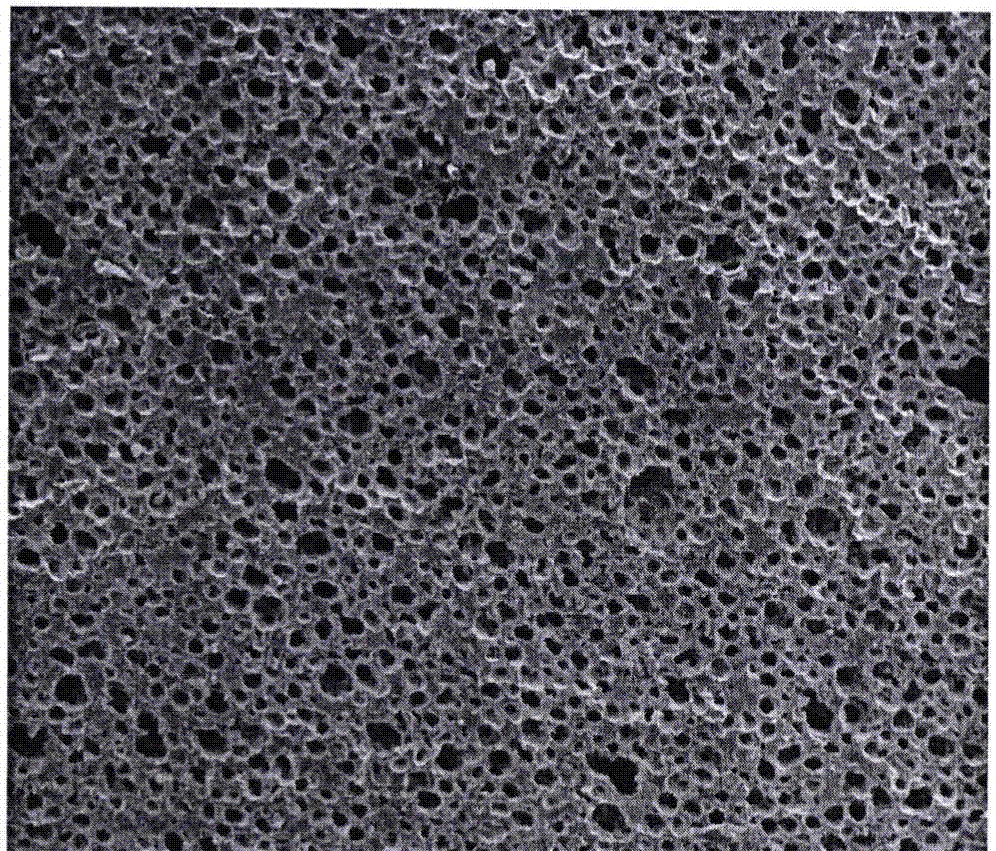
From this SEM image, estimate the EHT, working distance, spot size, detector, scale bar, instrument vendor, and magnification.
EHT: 15 kV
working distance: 17.1 mm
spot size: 3.5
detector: ETD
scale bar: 500000 nm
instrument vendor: FEI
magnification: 0.2 K X